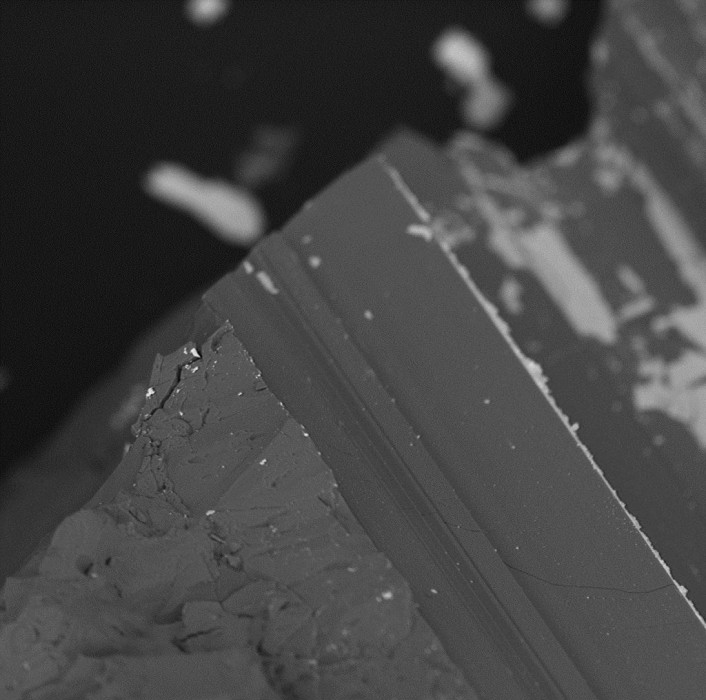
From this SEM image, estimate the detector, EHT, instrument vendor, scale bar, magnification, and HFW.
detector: SH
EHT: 5 kV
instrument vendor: other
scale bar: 30000 nm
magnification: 2.25 K X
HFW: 121 µm